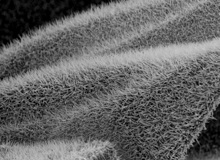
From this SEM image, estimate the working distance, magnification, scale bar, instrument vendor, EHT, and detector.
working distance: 9.5 mm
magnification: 3.5 K X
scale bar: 1000 nm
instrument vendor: JEOL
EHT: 10 kV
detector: SEI